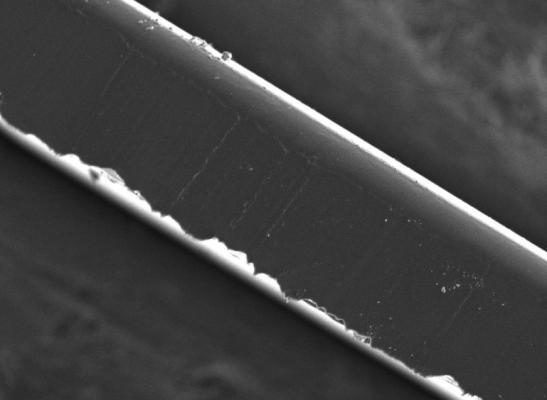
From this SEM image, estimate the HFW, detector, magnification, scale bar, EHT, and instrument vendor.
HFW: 252.55 µm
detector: SE Detector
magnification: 1.08 K X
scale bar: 100000 nm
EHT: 6 kV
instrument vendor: Tescan